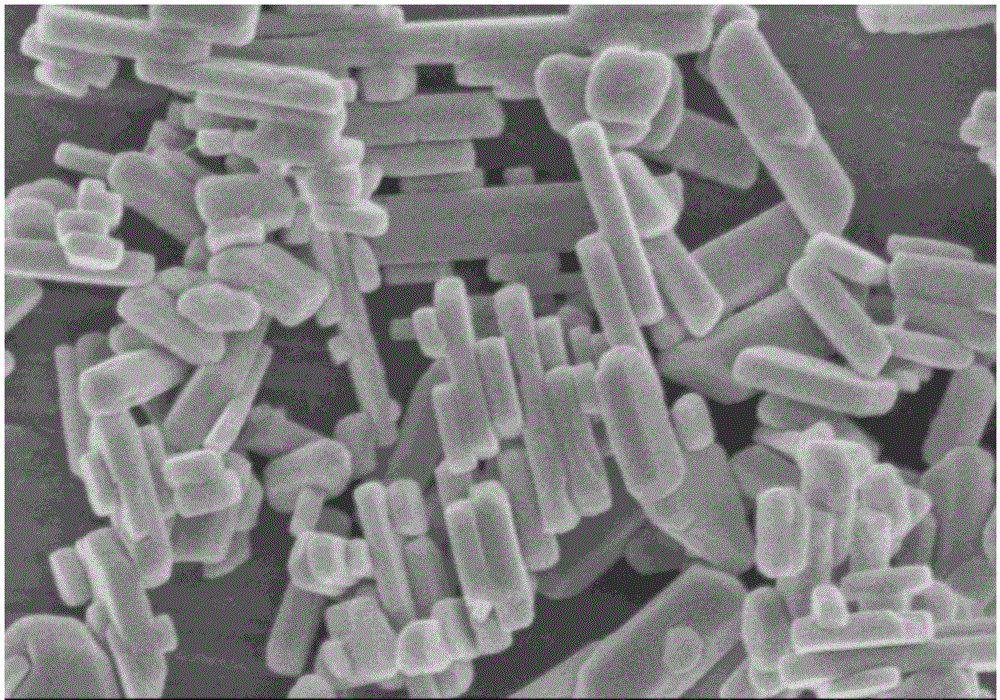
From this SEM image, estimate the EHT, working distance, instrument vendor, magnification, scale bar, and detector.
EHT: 3 kV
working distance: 10.2 mm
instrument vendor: Hitachi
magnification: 45 K X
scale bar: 1000 nm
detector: SE(M)